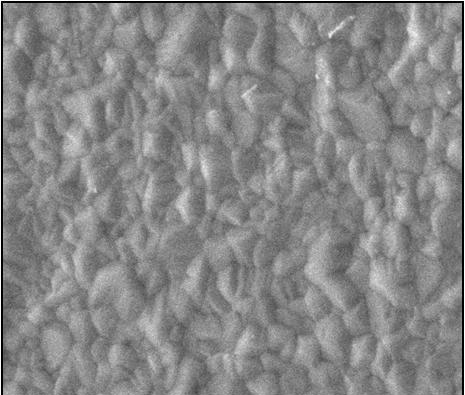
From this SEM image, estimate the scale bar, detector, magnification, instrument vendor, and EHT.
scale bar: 5000 nm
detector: SED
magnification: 5 K X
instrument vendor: other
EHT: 20 kV